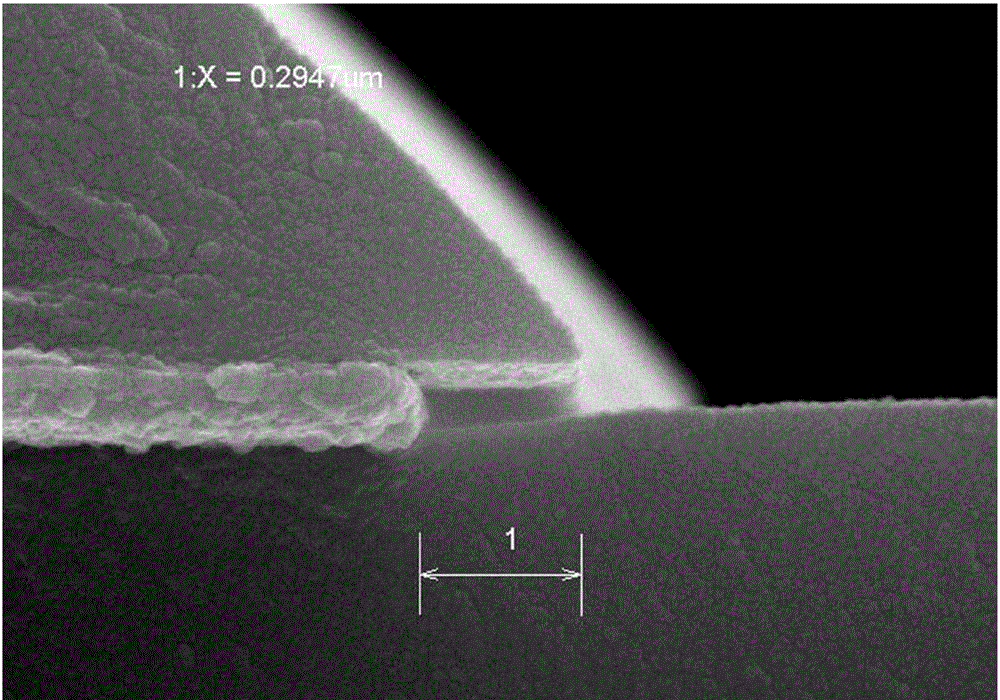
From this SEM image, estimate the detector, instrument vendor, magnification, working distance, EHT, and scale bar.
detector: SE(M)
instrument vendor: Hitachi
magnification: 70 K X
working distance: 7.4 mm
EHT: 15 kV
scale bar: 500 nm